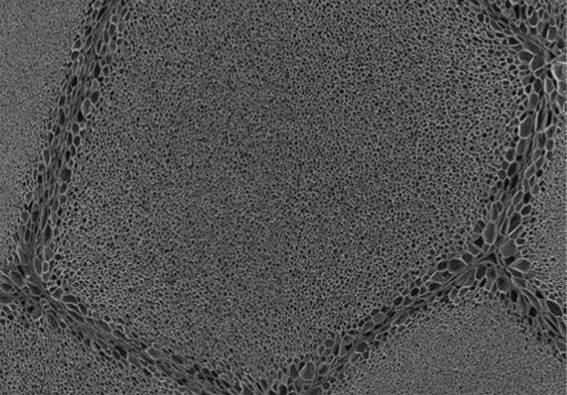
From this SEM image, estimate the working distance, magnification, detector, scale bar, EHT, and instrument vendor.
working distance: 42.2 mm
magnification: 0.015 K X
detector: SE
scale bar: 3000000 nm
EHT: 15 kV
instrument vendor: Hitachi